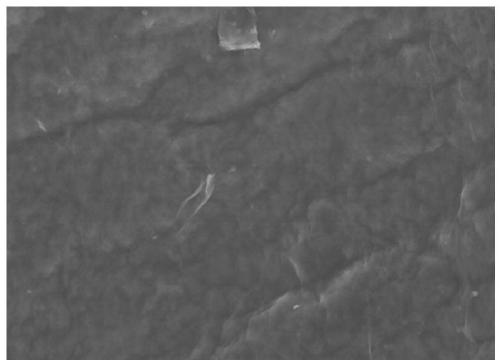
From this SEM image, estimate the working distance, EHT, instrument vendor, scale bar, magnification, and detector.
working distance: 9.1 mm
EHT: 5 kV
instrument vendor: Hitachi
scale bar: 5000 nm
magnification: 10 K X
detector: SE(M,LA0)